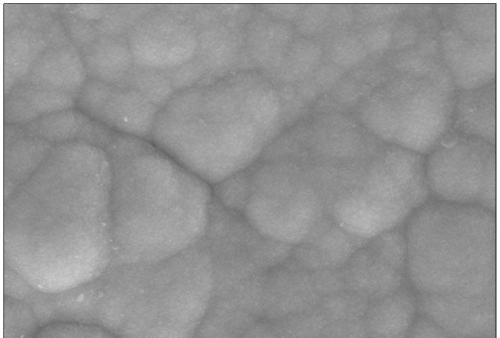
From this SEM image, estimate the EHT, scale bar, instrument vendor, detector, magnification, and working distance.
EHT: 10 kV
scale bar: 1000 nm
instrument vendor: Zeiss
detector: SE2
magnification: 10 K X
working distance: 18.8 mm